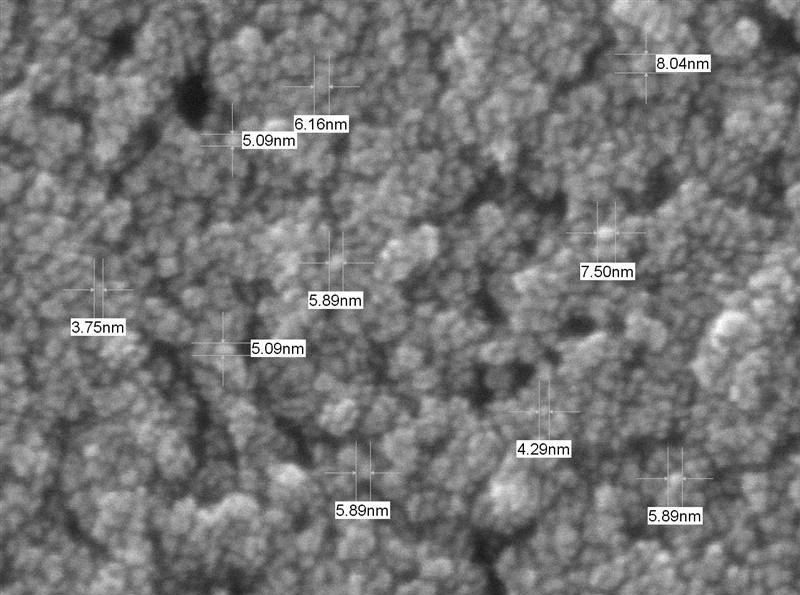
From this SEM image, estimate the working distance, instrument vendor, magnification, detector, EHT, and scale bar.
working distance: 4.5 mm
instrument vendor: JEOL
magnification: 350 K X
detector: SEI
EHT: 10 kV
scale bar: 10 nm